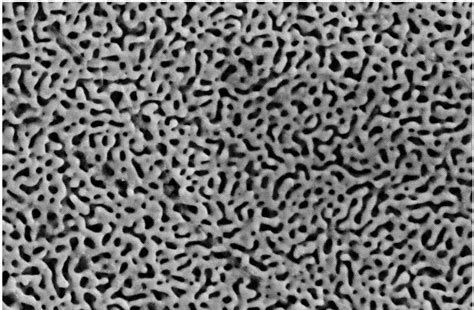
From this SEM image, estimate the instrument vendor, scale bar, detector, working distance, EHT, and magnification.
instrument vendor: Zeiss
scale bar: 200 nm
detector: SE2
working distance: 10 mm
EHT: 5 kV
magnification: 30 K X